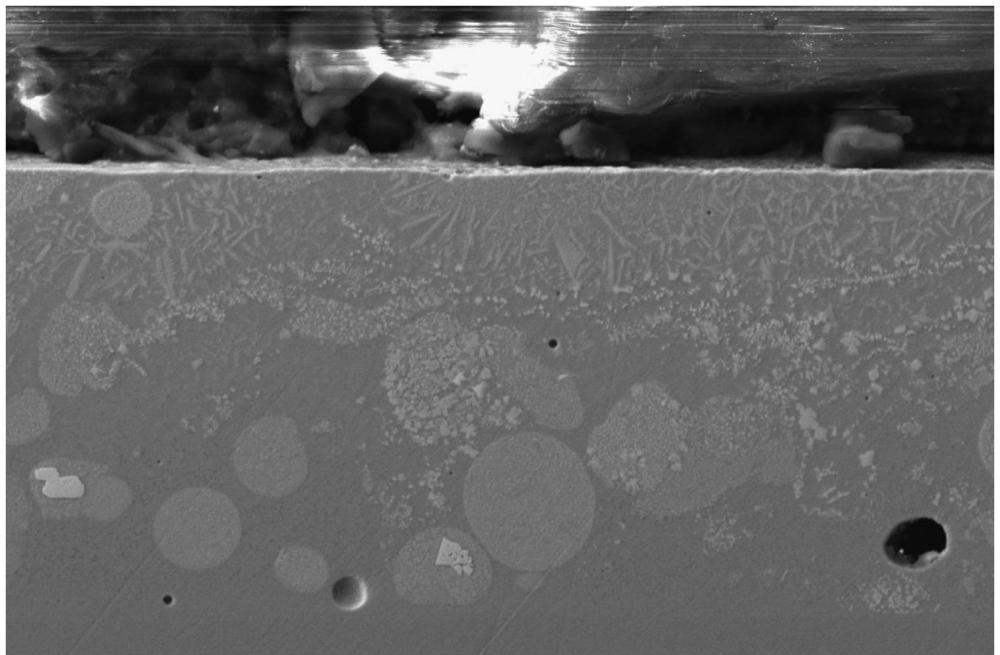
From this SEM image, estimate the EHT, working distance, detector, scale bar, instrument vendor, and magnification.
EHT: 20 kV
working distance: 10.2 mm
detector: ETD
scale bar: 50000 nm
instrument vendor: Thermo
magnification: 2 K X